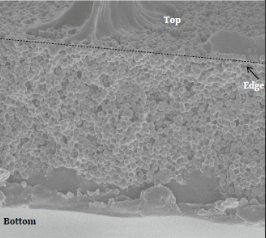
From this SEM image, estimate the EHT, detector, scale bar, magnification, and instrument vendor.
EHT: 5 kV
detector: SEI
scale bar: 10000 nm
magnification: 2.7 K X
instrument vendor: JEOL